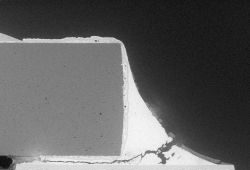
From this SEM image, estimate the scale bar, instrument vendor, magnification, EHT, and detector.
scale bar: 400000 nm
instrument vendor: Hitachi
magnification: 0.12 K X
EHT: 5 kV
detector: BSECOMP